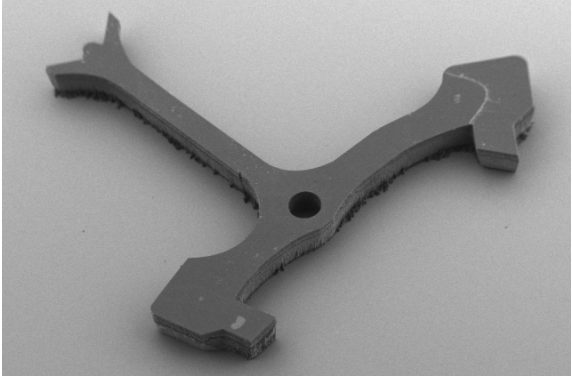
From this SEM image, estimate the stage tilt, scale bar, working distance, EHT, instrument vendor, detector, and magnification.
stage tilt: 40°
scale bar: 100000 nm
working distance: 7.3 mm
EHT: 3 kV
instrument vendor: Zeiss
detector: HE-SE2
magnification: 0.044 K X